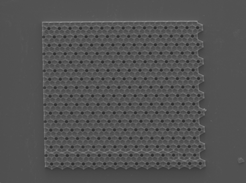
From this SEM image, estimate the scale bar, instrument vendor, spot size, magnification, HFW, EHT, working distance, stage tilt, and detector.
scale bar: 200000 nm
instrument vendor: FEI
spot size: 4.5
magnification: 0.5 K X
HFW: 597 µm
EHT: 15 kV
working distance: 8.4 mm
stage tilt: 0°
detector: ETD